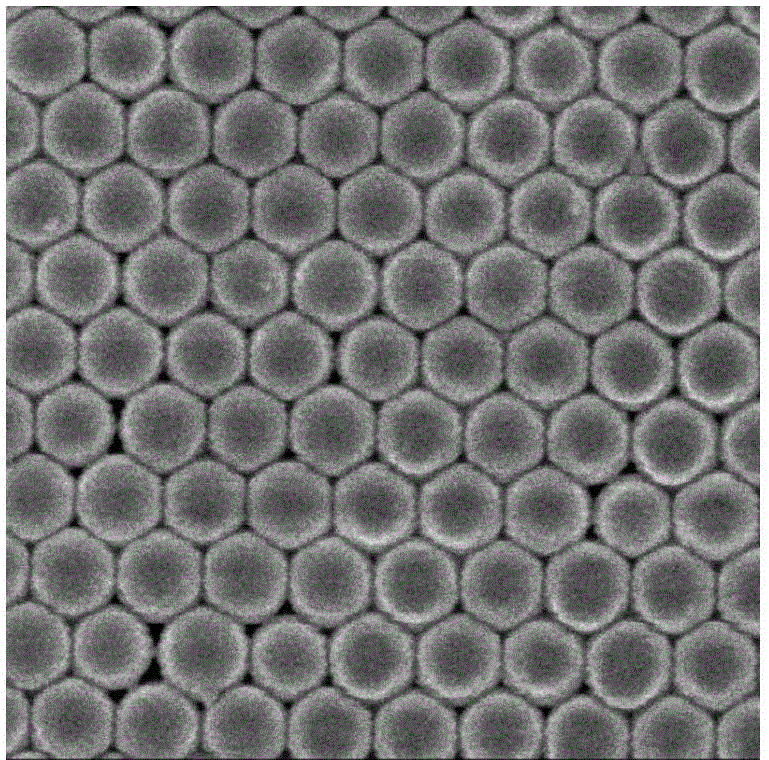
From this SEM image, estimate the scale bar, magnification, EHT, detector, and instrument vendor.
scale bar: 100 nm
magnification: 30 K X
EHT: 3 kV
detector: SEI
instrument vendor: JEOL